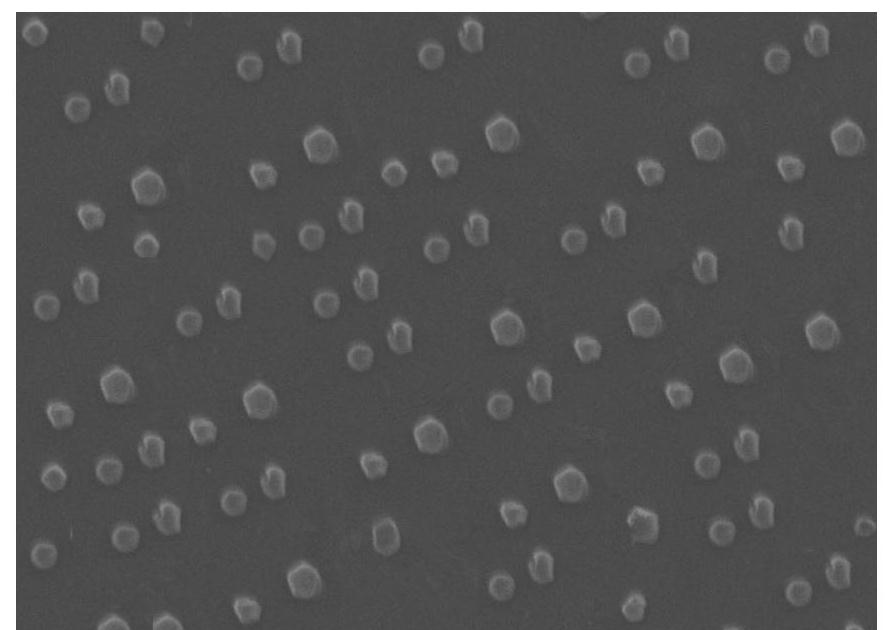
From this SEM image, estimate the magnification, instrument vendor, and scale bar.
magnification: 10 K X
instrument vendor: JEOL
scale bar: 1000 nm